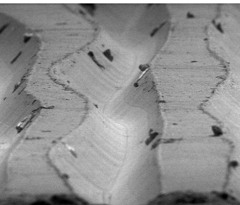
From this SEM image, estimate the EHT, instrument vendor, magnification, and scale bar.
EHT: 5 kV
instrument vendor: JEOL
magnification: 0.48 K X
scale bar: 50000 nm